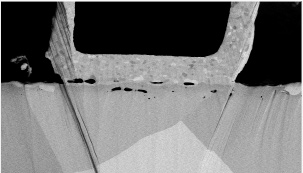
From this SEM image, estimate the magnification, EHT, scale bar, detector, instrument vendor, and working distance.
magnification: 1 K X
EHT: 5 kV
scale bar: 50000 nm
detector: PDBSE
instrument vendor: Hitachi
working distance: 7.8 mm